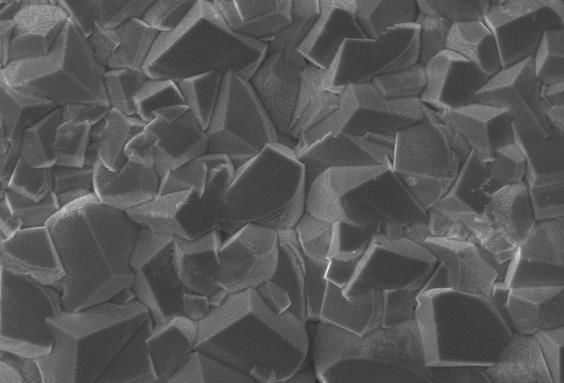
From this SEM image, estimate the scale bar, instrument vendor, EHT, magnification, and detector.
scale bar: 10000 nm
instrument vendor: JEOL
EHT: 15 kV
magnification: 1 K X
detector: SEI